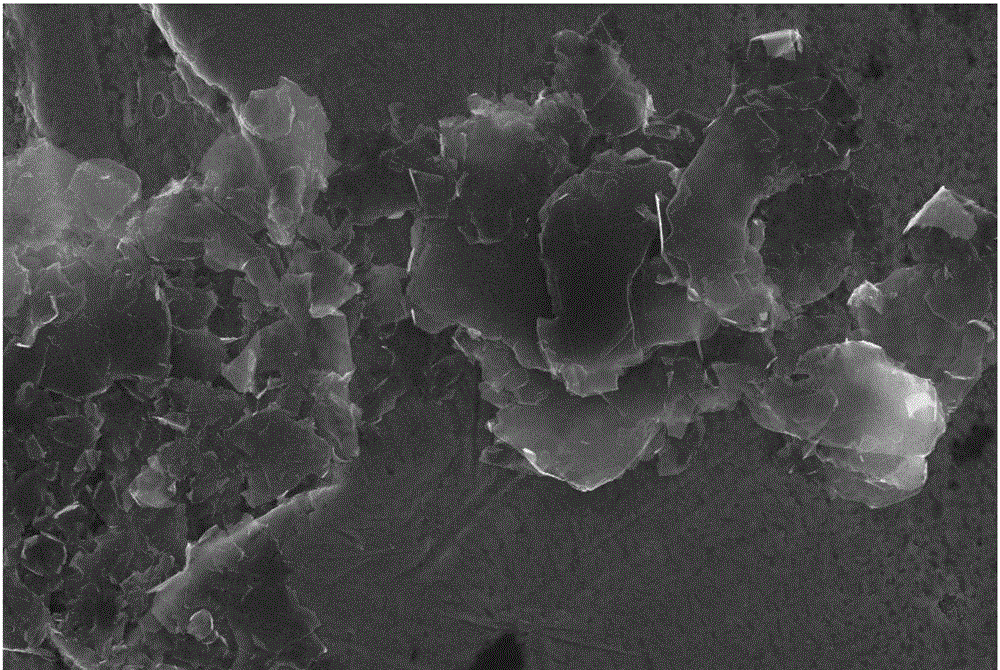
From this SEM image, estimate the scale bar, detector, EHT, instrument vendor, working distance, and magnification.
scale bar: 2000 nm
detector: InlensDuo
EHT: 15 kV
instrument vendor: Zeiss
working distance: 7 mm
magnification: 3 K X